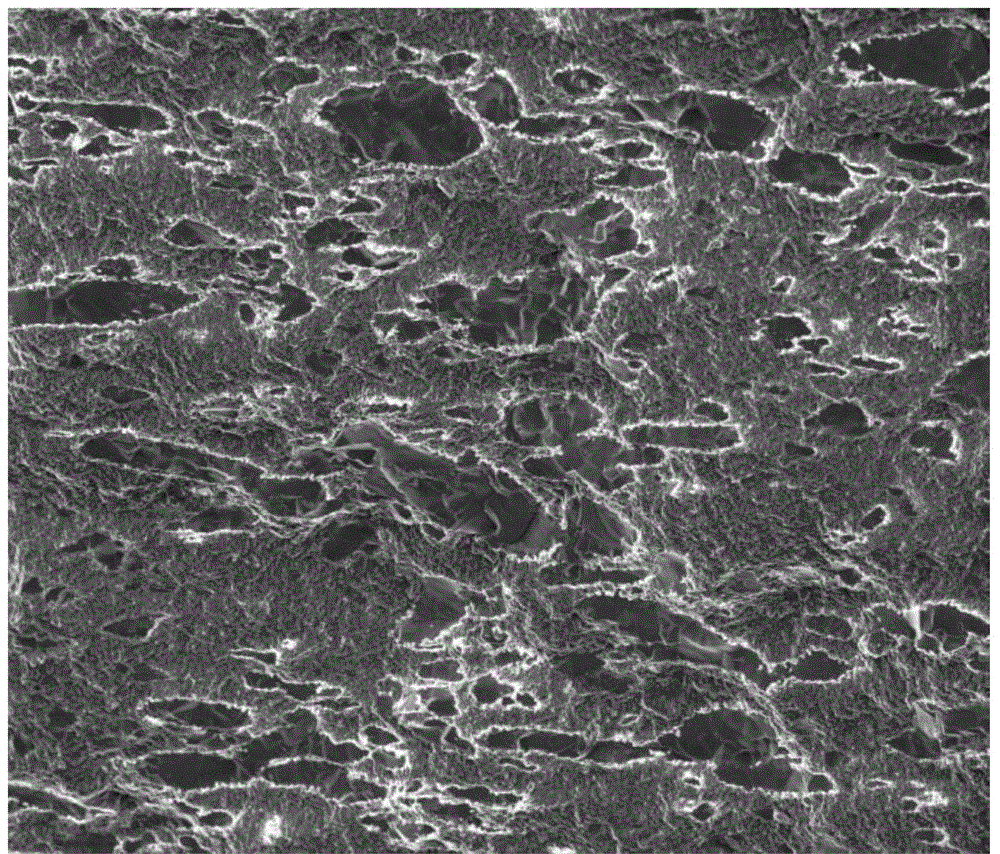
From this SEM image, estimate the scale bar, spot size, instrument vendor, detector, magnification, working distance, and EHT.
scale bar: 50000 nm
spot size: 3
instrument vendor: FEI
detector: ETD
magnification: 2 K X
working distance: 11.2 mm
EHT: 5 kV